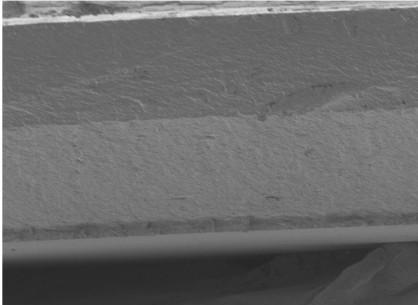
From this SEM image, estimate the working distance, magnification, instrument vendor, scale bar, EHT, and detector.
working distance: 8 mm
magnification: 0.45 K X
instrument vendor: JEOL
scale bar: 10000 nm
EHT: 3 kV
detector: LEI